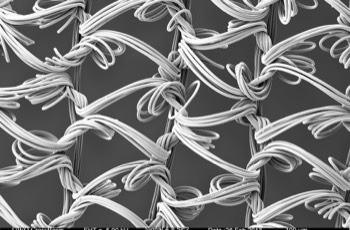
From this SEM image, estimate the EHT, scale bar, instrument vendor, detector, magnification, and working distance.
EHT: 5 kV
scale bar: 100000 nm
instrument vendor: Zeiss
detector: SE2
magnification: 0.05 K X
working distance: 19.4 mm